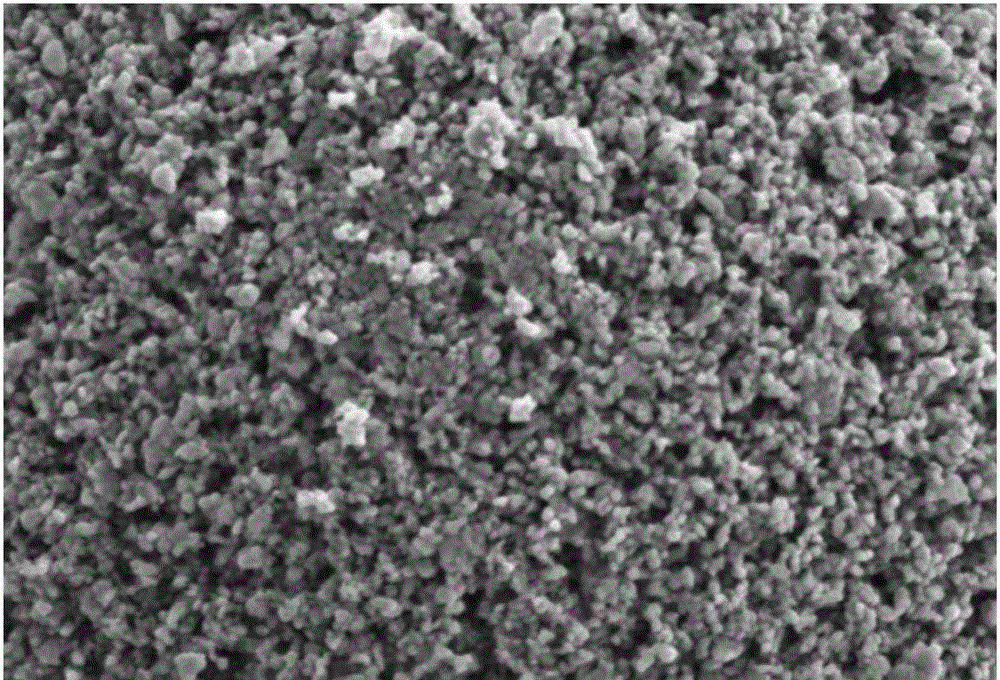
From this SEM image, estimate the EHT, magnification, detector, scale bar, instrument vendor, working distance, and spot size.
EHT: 5 kV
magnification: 10 K X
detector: SEI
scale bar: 1000 nm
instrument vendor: JEOL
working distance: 10 mm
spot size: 30